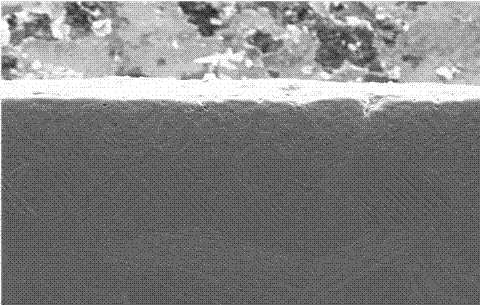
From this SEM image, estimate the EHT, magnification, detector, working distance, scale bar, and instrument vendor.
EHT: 20 kV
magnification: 0.35 K X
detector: LM(UL)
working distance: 11.1 mm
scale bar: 100000 nm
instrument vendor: Hitachi